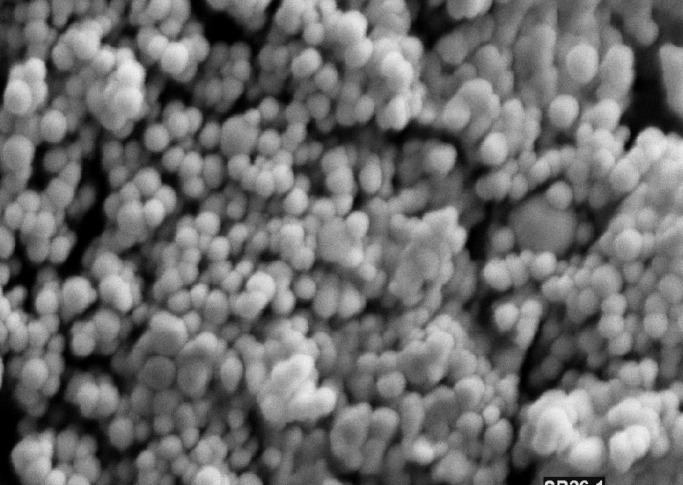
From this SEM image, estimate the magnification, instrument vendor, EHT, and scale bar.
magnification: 15 K X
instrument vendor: other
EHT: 25 kV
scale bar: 1000 nm